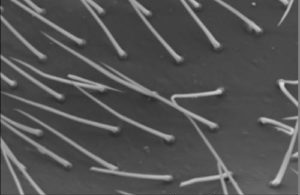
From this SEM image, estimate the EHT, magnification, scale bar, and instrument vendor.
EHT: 20 kV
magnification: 0.35 K X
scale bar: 50000 nm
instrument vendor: JEOL